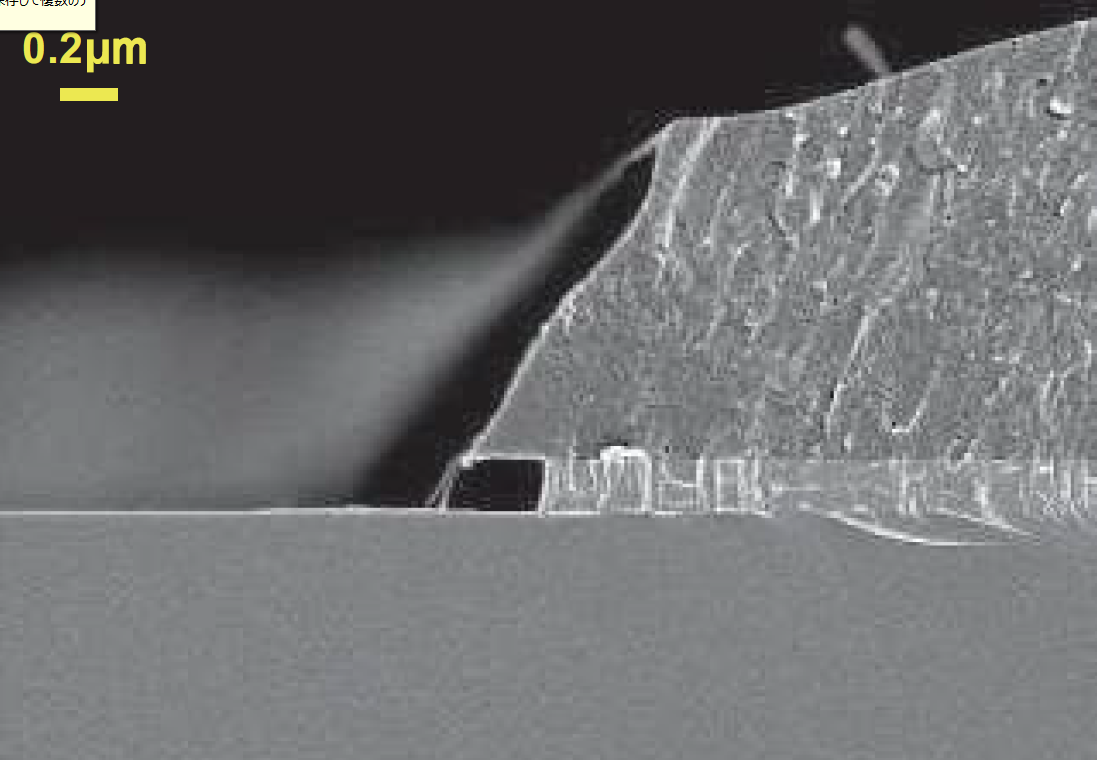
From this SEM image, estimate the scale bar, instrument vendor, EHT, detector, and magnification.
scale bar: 2000 nm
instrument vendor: Hitachi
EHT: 1.5 kV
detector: SE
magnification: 20 K X